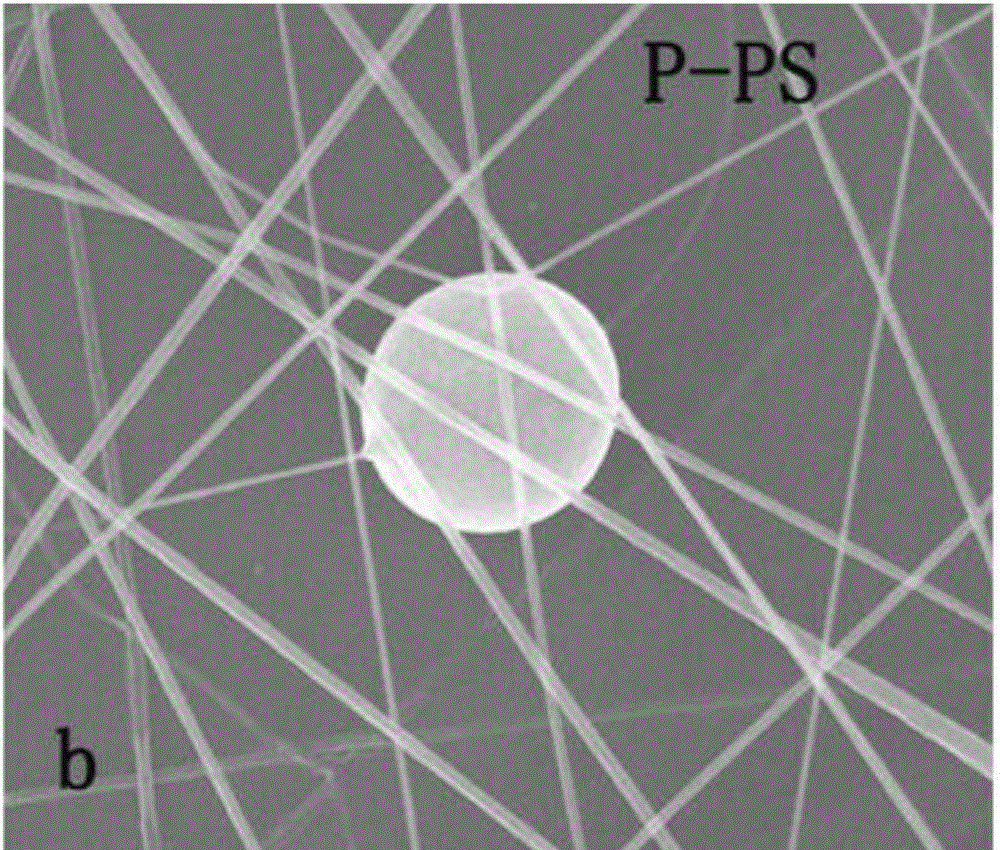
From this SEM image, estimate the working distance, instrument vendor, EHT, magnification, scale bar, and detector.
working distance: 10.8 mm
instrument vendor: FEI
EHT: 20 kV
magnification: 10 K X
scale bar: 10000 nm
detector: ETD